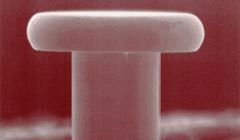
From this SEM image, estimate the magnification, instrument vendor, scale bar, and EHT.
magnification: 0.15 K X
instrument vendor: JEOL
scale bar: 100000 nm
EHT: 15 kV